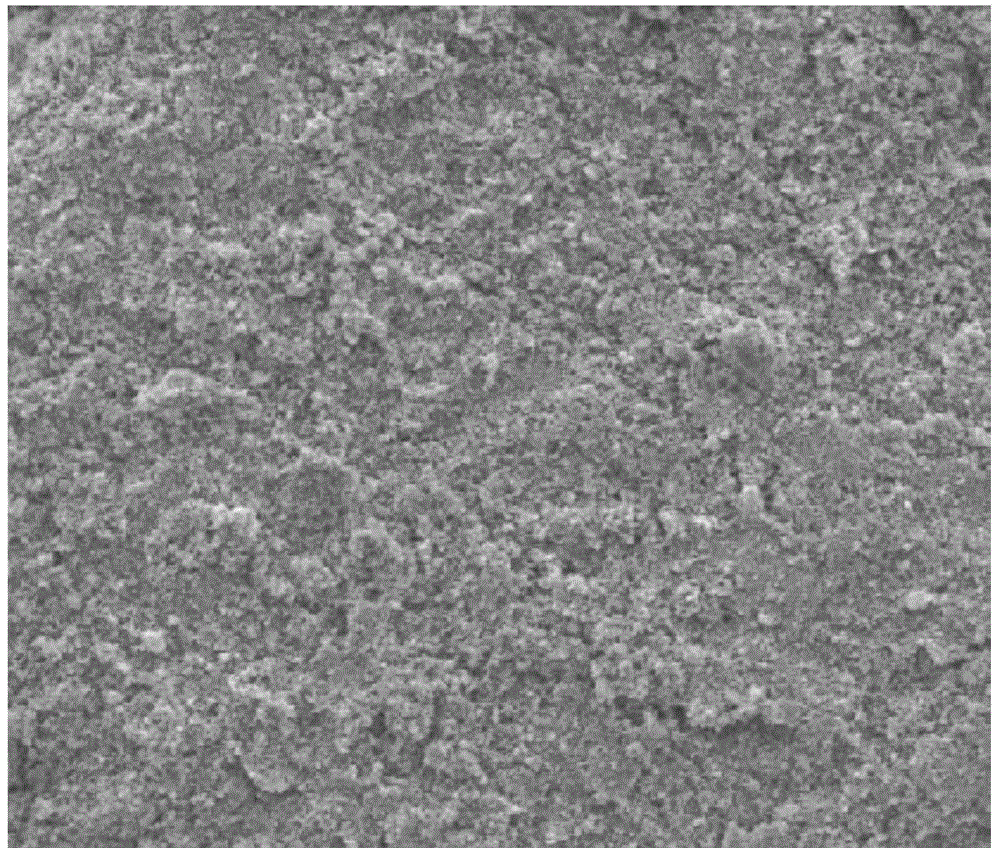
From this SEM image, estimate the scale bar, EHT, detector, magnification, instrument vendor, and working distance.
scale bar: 50000 nm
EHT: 15 kV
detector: SE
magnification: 1 K X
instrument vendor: FEI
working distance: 17.6 mm